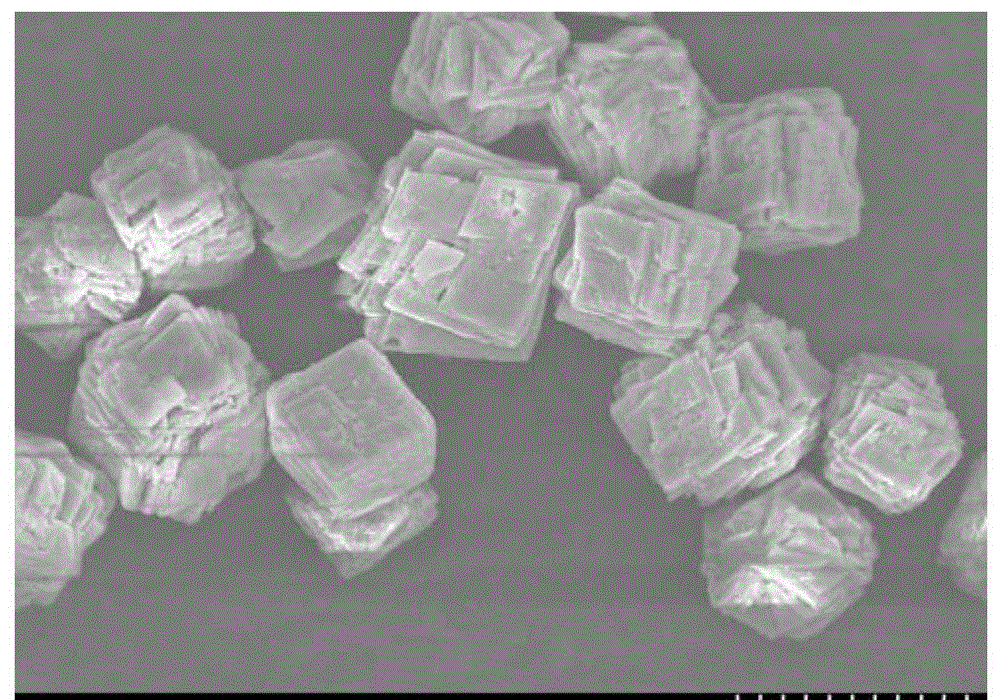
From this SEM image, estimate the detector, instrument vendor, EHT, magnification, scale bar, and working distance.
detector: SE(M)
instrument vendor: Hitachi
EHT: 10 kV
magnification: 3 K X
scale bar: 10000 nm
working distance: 9.1 mm